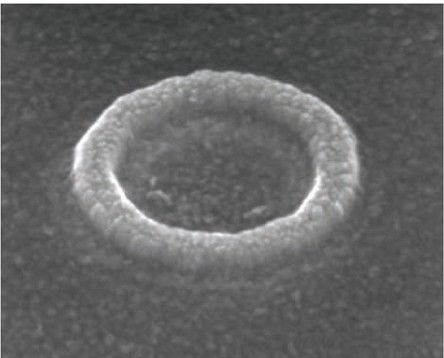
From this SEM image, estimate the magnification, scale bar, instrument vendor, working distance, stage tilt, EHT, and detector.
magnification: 380.339 K X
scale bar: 200 nm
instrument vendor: FEI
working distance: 6.4 mm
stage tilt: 45°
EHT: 10 kV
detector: TLD